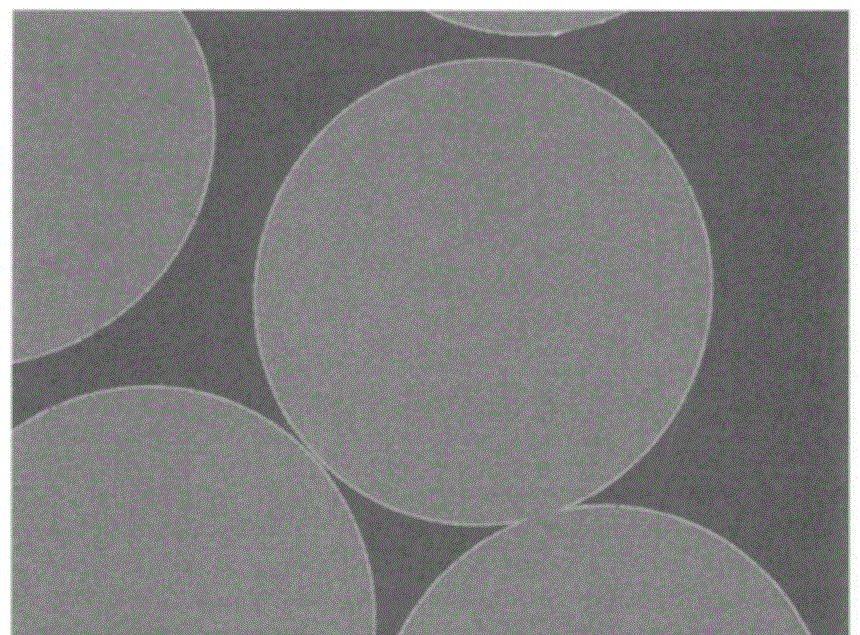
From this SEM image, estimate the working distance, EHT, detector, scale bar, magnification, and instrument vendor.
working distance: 8 mm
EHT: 5 kV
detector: LABE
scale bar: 1000 nm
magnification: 5 K X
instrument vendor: JEOL